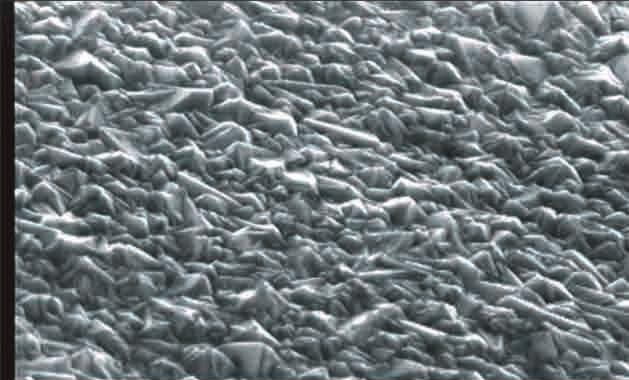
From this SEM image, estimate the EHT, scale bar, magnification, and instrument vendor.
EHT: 15 kV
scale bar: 10000 nm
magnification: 2 K X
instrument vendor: JEOL